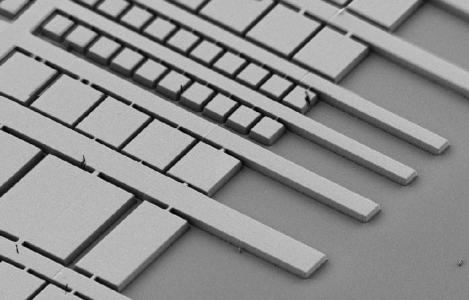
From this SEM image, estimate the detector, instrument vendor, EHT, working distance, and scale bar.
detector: SE2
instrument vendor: Zeiss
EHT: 2 kV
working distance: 8 mm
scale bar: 10000 nm